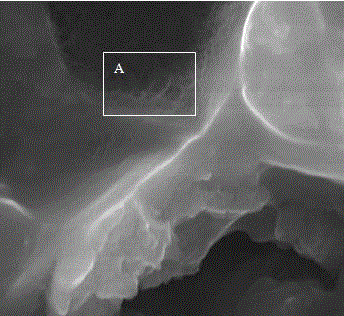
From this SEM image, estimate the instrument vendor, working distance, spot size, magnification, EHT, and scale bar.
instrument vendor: FEI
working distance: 5.7 mm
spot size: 2.5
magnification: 50 K X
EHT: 20 kV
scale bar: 1000 nm